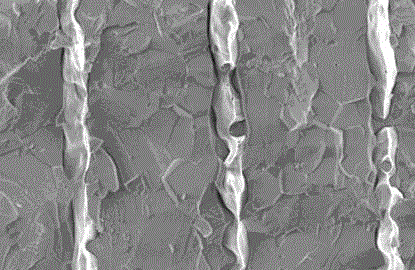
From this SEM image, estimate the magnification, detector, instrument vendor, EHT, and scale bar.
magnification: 8 K X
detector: SEI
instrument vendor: JEOL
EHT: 20 kV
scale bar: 2000 nm